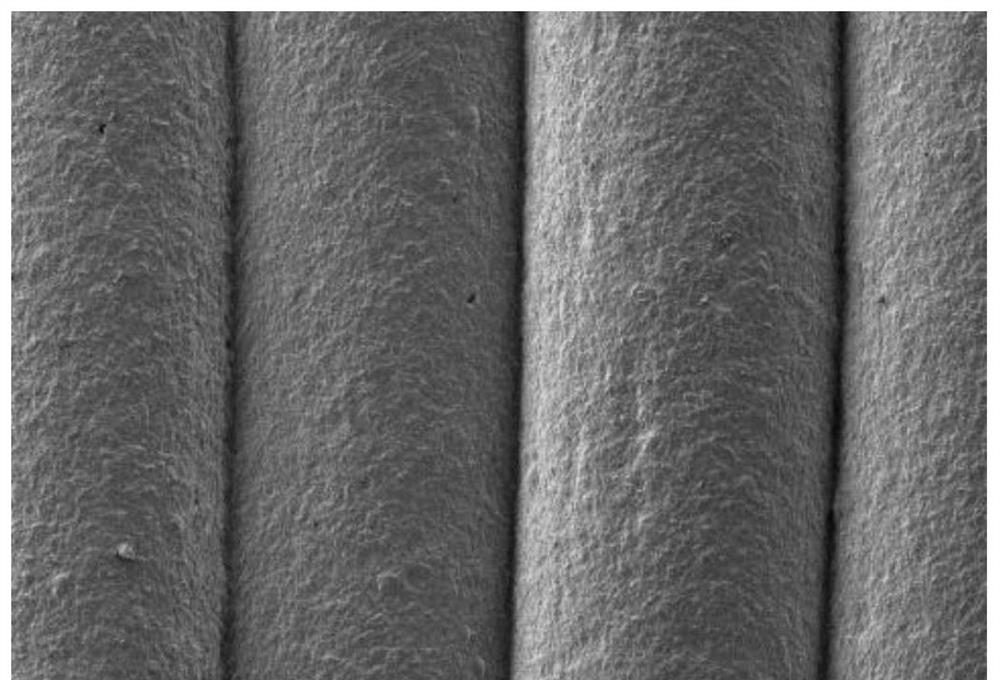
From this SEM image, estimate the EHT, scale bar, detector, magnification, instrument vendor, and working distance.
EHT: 5 kV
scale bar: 1000000 nm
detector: LM(L)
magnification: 0.05 K X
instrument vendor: Hitachi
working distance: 8.5 mm